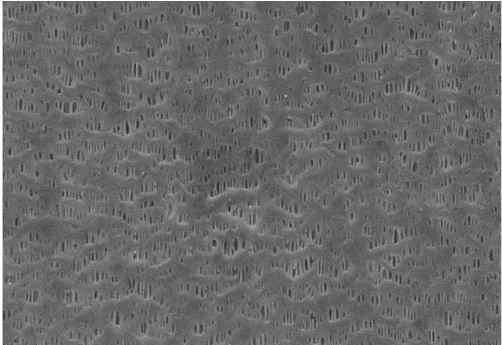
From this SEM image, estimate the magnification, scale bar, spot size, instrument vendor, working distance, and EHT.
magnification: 30 K X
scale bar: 1000 nm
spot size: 7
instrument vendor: JEOL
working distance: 5 mm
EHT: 20 kV